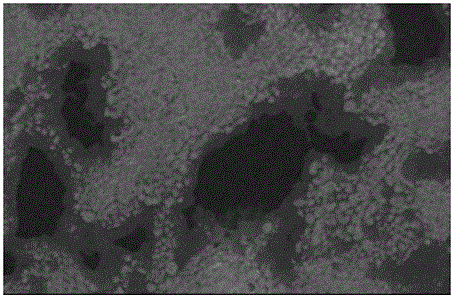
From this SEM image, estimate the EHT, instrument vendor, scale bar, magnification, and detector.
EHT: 20 kV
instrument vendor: JEOL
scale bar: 10000 nm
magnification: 1 K X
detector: SEI